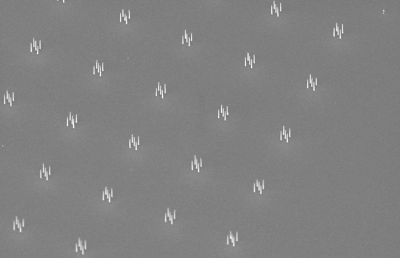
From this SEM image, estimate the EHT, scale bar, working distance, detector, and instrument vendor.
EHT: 15 kV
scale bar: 2000 nm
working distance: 6.5 mm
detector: SE1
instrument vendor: Zeiss